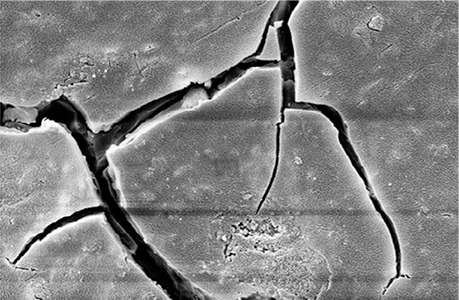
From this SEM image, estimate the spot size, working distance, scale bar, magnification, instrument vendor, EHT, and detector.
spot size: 300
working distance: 12.38 mm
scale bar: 2000 nm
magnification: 10 K X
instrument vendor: Zeiss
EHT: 10 kV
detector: SE1